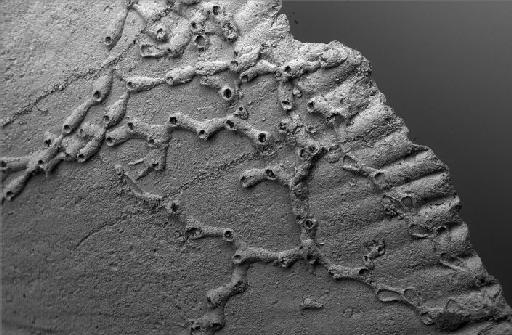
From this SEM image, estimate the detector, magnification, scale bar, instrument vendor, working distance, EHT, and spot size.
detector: BSD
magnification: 0.05 K X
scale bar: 200000 nm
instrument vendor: Zeiss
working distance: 12 mm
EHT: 20 kV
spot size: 500